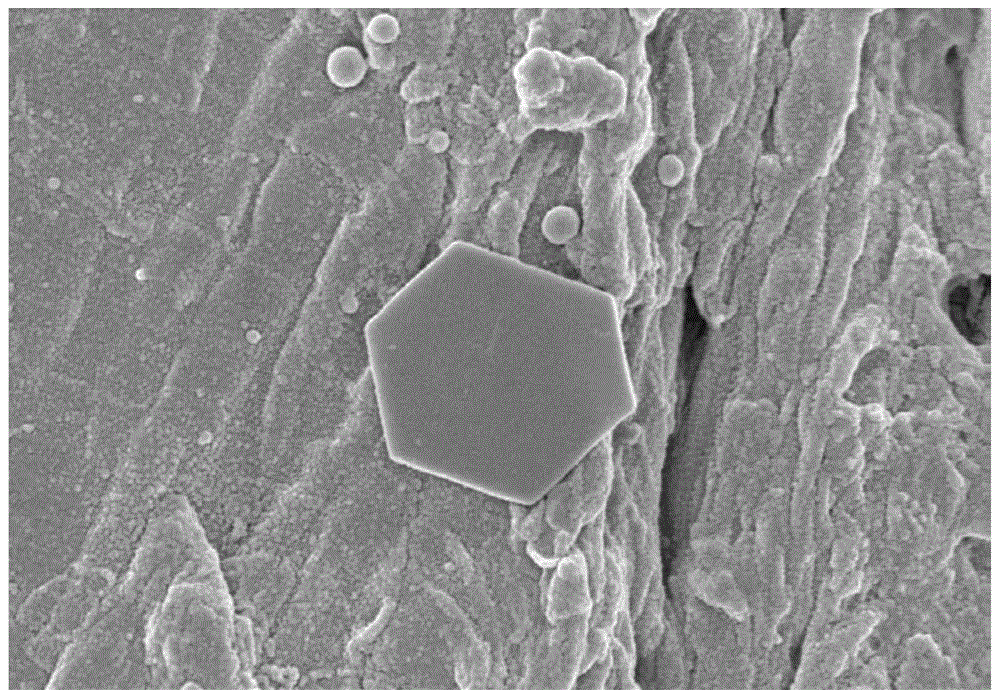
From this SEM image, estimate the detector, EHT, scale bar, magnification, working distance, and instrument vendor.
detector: SE(TUL)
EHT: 3 kV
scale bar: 2000 nm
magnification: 20 K X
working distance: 4.9 mm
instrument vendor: Hitachi